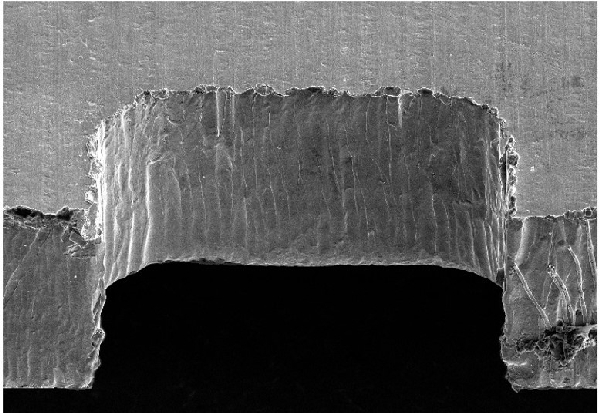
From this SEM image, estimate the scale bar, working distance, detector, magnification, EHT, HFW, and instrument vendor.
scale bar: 100000 nm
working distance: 7.7 mm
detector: ETD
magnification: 0.35 K X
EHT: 10 kV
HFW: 363 µm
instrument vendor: FEI